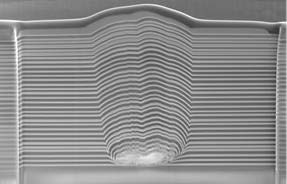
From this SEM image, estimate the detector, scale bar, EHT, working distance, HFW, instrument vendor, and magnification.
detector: ETD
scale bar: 5000 nm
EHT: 5 kV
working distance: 4.1 mm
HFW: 17.3 µm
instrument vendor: FEI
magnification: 12 K X